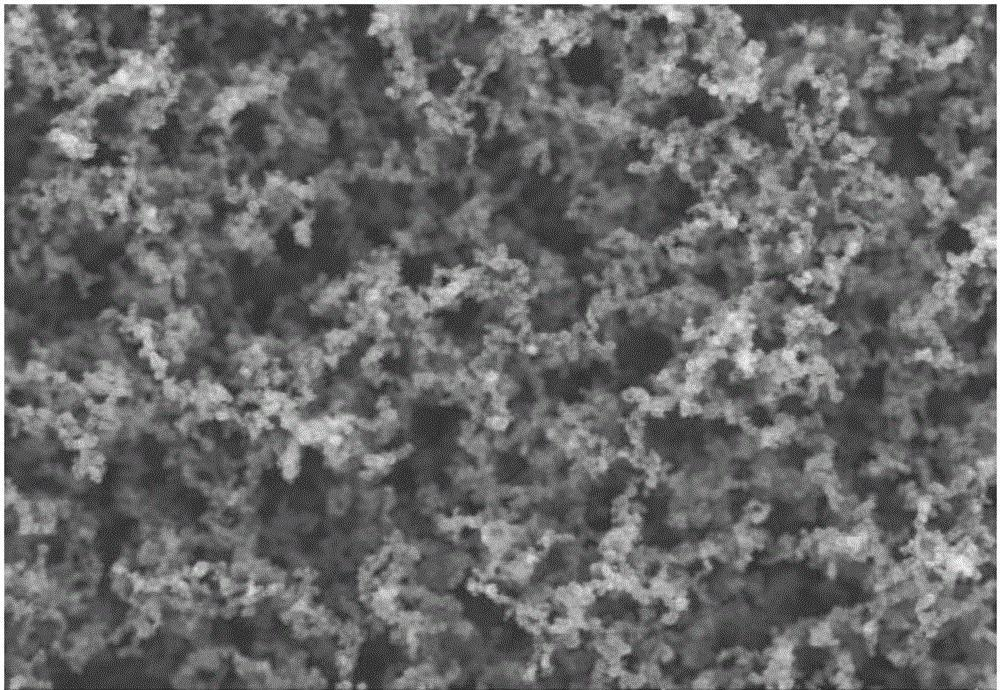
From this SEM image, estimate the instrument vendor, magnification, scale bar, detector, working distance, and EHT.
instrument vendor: Hitachi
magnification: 20 K X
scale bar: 2000 nm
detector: SE(UL)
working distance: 5.6 mm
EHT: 10 kV